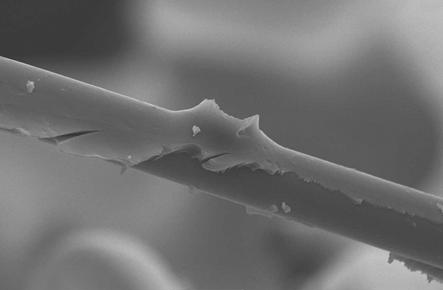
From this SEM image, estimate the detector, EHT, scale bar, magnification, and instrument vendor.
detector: SEI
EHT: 15 kV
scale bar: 10000 nm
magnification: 2 K X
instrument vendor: JEOL